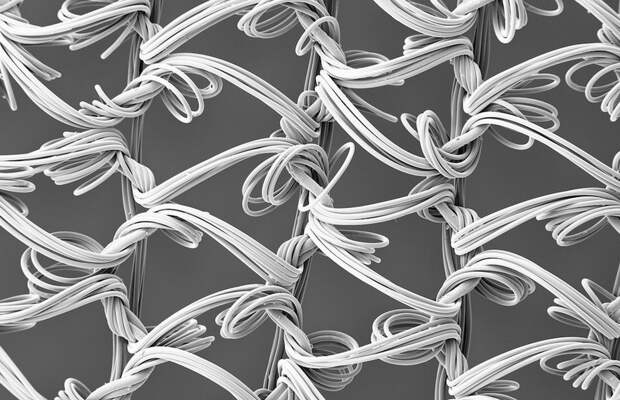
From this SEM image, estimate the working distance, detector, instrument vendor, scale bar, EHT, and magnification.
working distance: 19.4 mm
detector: SE2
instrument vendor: Zeiss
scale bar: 100000 nm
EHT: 5 kV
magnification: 0.05 K X